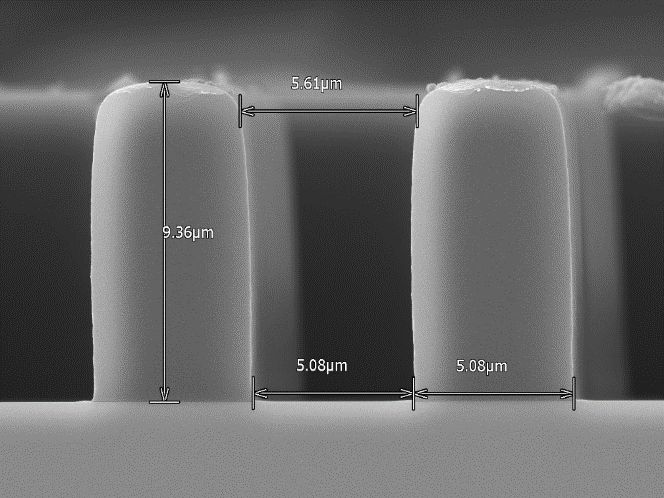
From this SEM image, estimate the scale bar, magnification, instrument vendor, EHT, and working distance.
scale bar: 5000 nm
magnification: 6 K X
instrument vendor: Hitachi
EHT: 10 kV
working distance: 15.6 mm